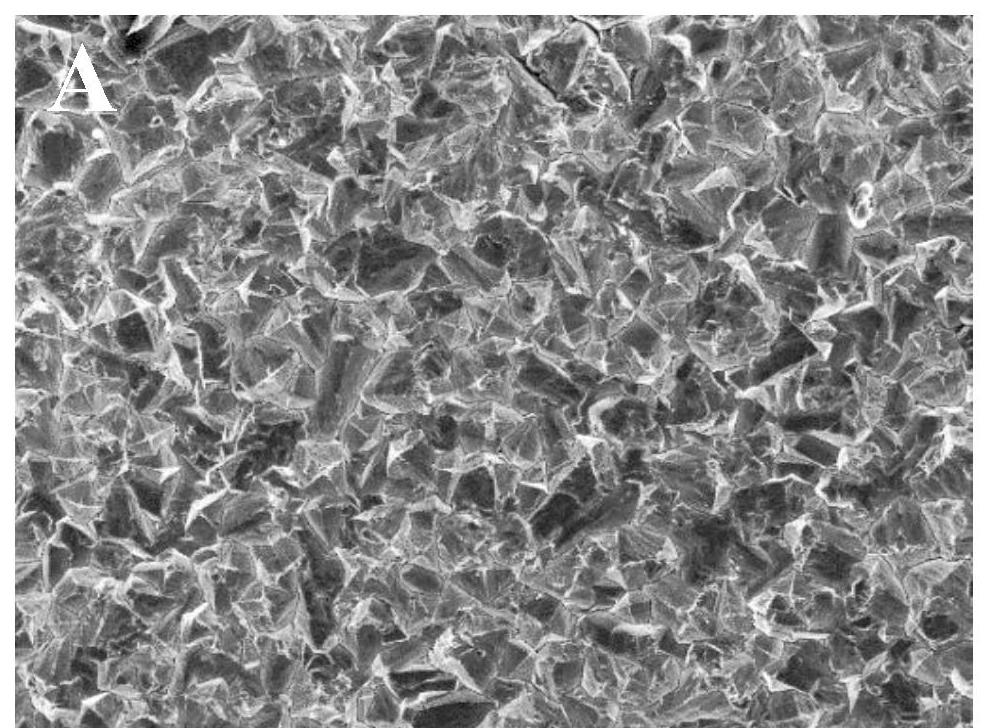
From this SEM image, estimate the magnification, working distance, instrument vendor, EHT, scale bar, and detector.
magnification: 1 K X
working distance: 7.6 mm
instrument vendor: JEOL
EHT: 10 kV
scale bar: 10000 nm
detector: SEI